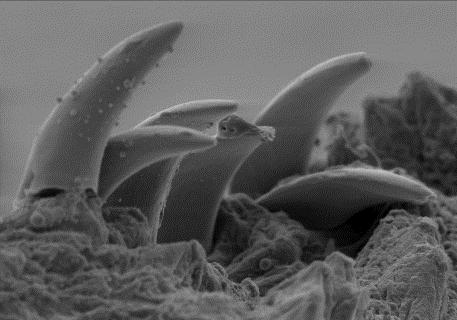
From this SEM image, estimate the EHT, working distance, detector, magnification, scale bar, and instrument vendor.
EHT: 10 kV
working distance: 9 mm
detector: SE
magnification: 0.49 K X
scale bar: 20000 nm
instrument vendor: other